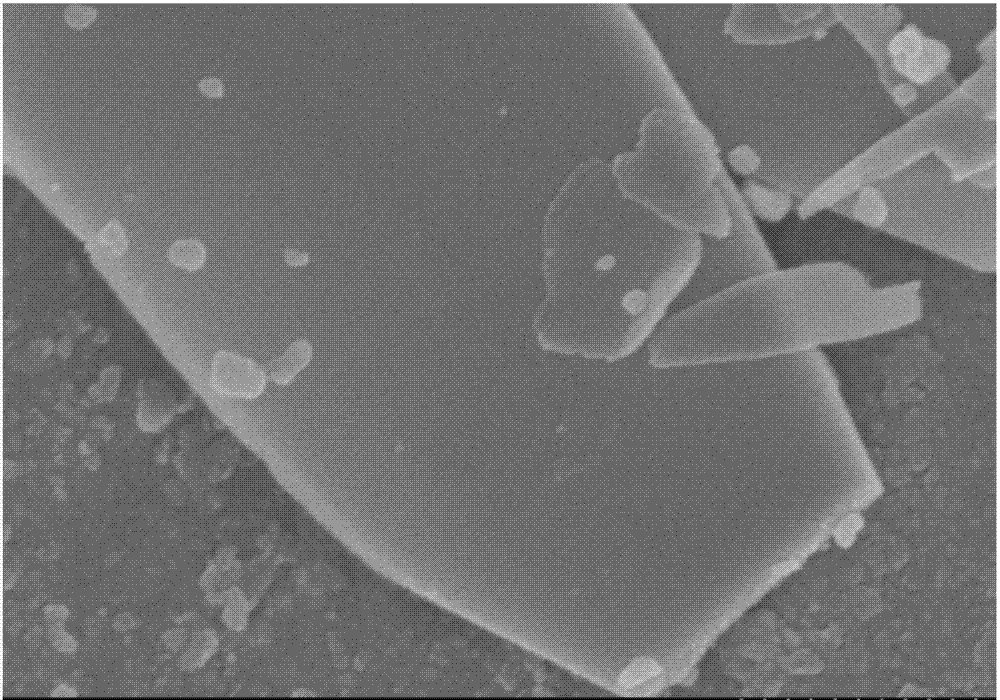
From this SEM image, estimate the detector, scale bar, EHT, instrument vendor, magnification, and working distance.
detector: SE(M)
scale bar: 500 nm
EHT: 3 kV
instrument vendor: Hitachi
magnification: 60 K X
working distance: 8.8 mm